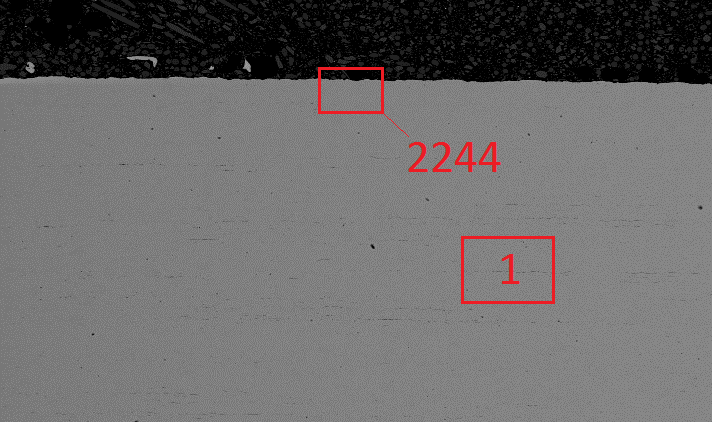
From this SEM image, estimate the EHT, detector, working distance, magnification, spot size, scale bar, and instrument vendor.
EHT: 20 kV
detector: BSE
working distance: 13.7 mm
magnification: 0.05 K X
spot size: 4.6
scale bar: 500000 nm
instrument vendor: FEI